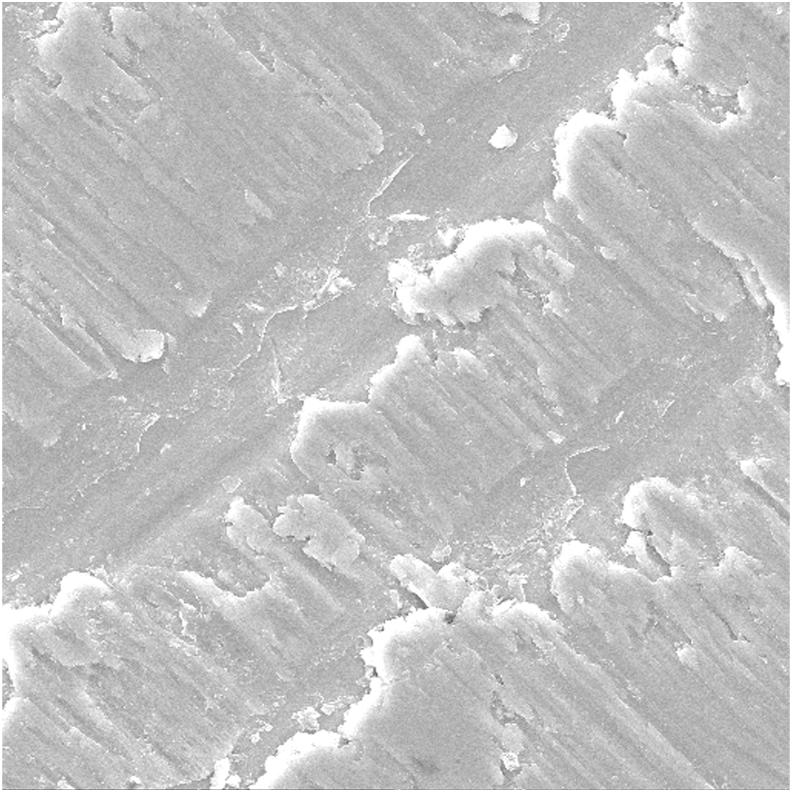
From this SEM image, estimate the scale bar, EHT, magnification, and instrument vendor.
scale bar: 20000 nm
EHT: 30 kV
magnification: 1 K X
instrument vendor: Tescan